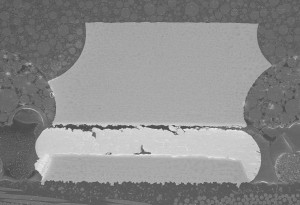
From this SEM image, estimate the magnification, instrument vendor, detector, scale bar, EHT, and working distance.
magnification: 0.3 K X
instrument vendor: Hitachi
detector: SE(UL)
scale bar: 100000 nm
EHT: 10 kV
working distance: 14.9 mm